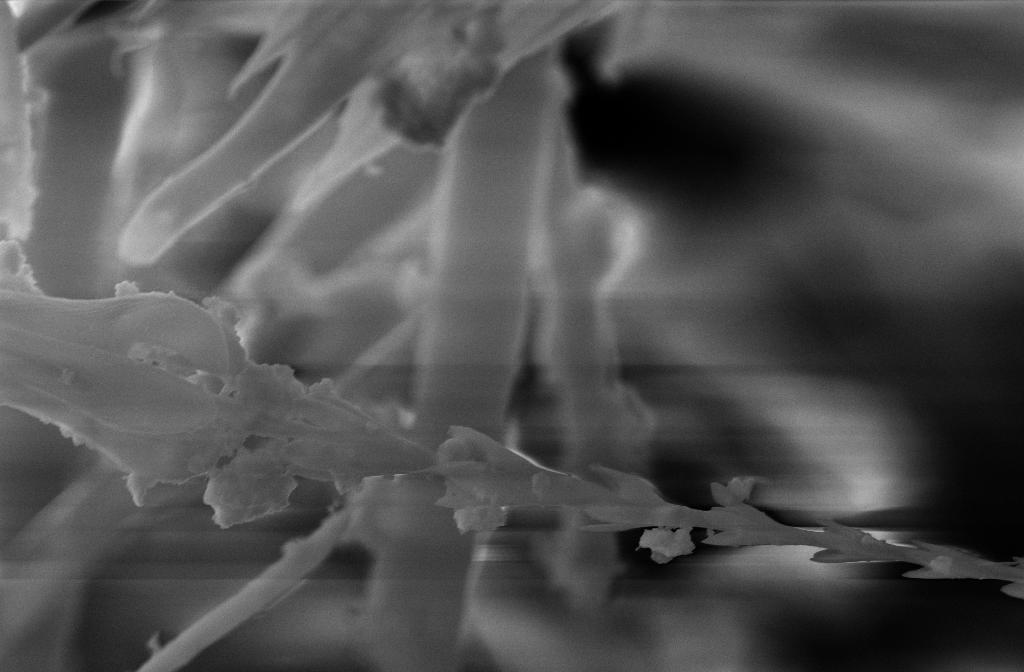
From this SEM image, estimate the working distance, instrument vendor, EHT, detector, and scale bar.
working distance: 15 mm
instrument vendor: Zeiss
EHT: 20 kV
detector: SE2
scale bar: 2000 nm